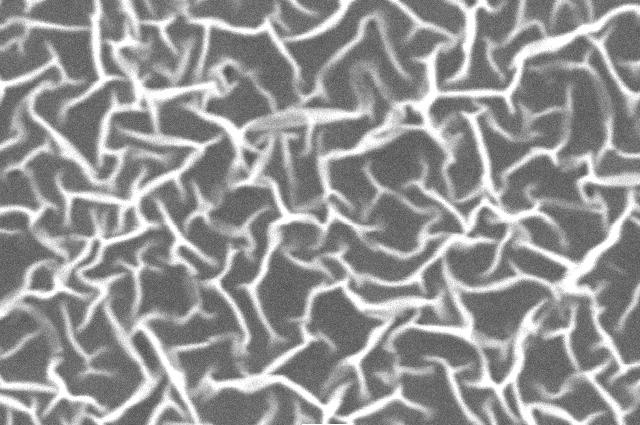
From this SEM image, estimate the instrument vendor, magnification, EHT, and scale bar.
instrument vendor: JEOL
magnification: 10 K X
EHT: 10 kV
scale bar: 1000 nm